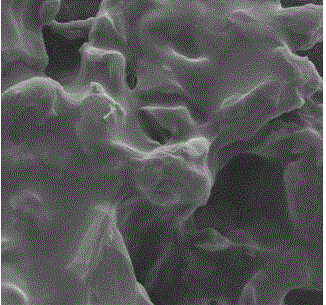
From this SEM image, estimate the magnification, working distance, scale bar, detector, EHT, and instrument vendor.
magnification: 12.19 K X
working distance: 12.7 mm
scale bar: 1000 nm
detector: SE2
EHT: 5 kV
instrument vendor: Zeiss